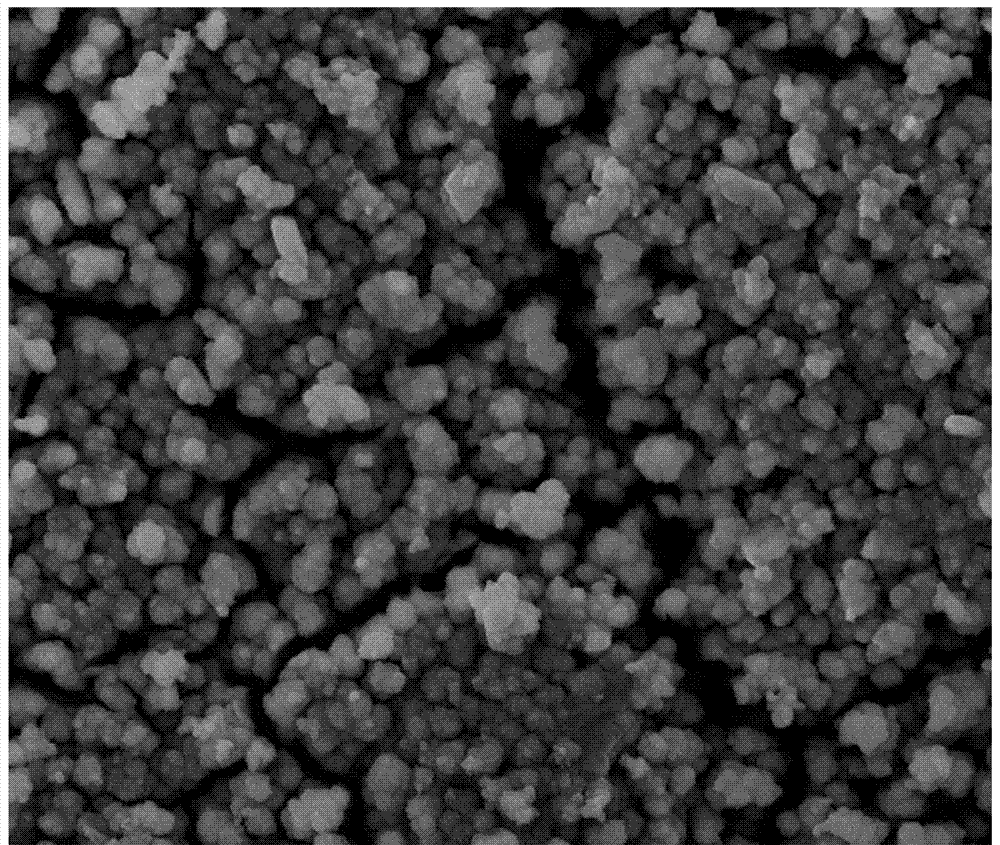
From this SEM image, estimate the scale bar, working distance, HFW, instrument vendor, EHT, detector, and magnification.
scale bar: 10000 nm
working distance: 8.8 mm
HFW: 60.3 µm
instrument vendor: Tescan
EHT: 15 kV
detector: SE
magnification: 2.5 K X